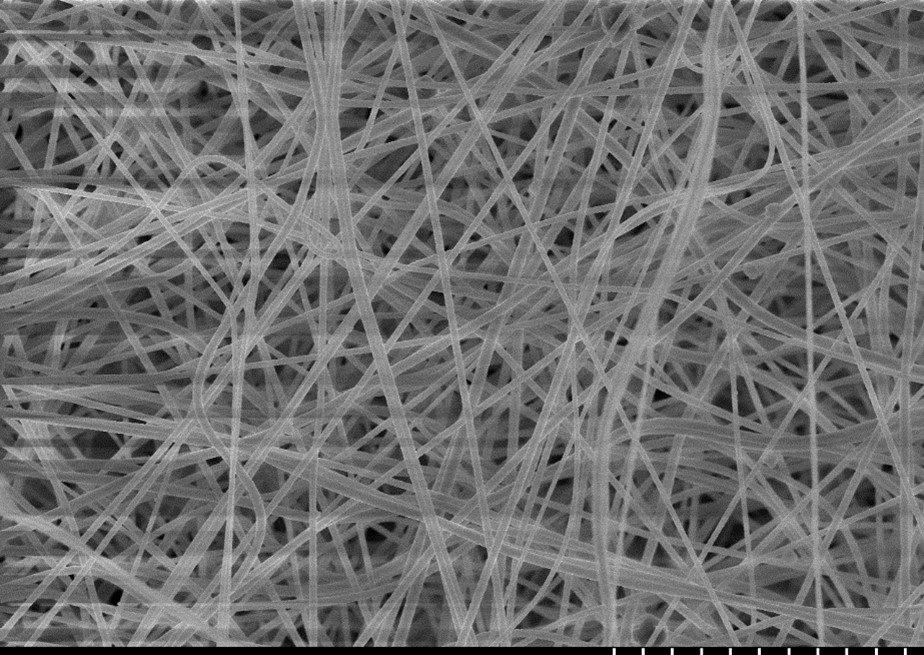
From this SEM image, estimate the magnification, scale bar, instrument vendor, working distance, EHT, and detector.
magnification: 2 K X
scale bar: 20000 nm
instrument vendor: Hitachi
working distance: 8.4 mm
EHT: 10 kV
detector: SE(M)